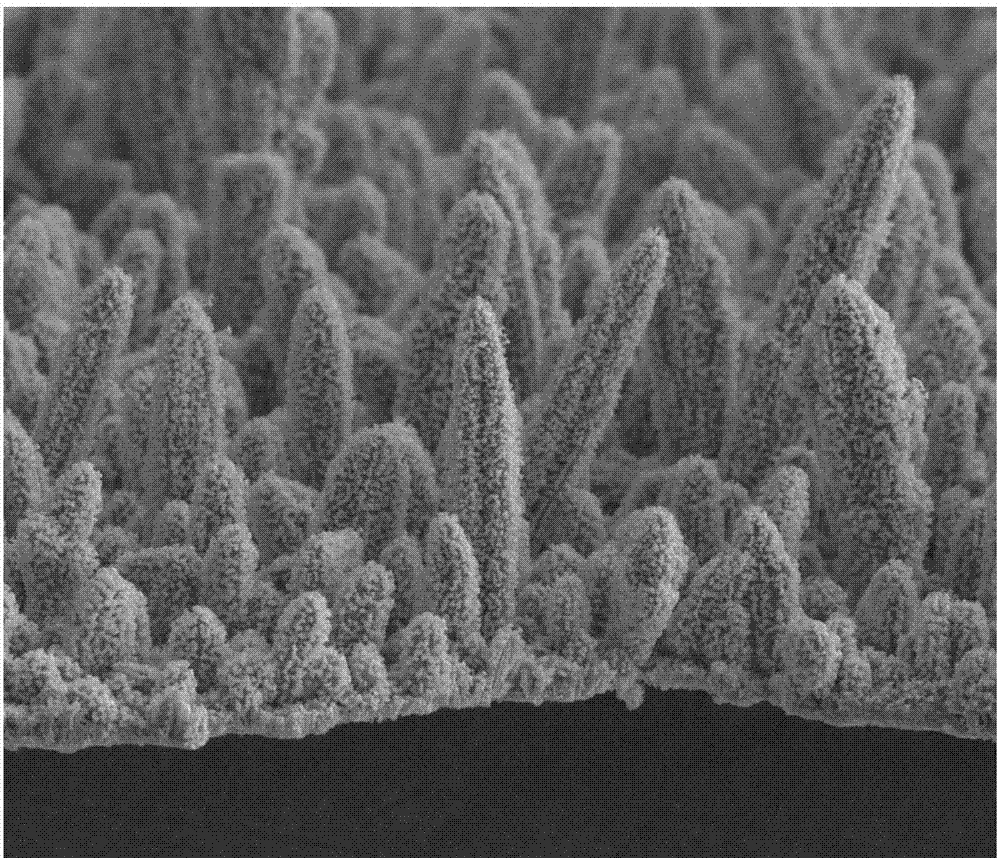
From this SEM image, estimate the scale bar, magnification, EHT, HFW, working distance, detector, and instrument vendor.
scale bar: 20000 nm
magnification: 1 K X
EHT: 20 kV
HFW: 140 µm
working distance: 12 mm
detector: ETD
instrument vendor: FEI